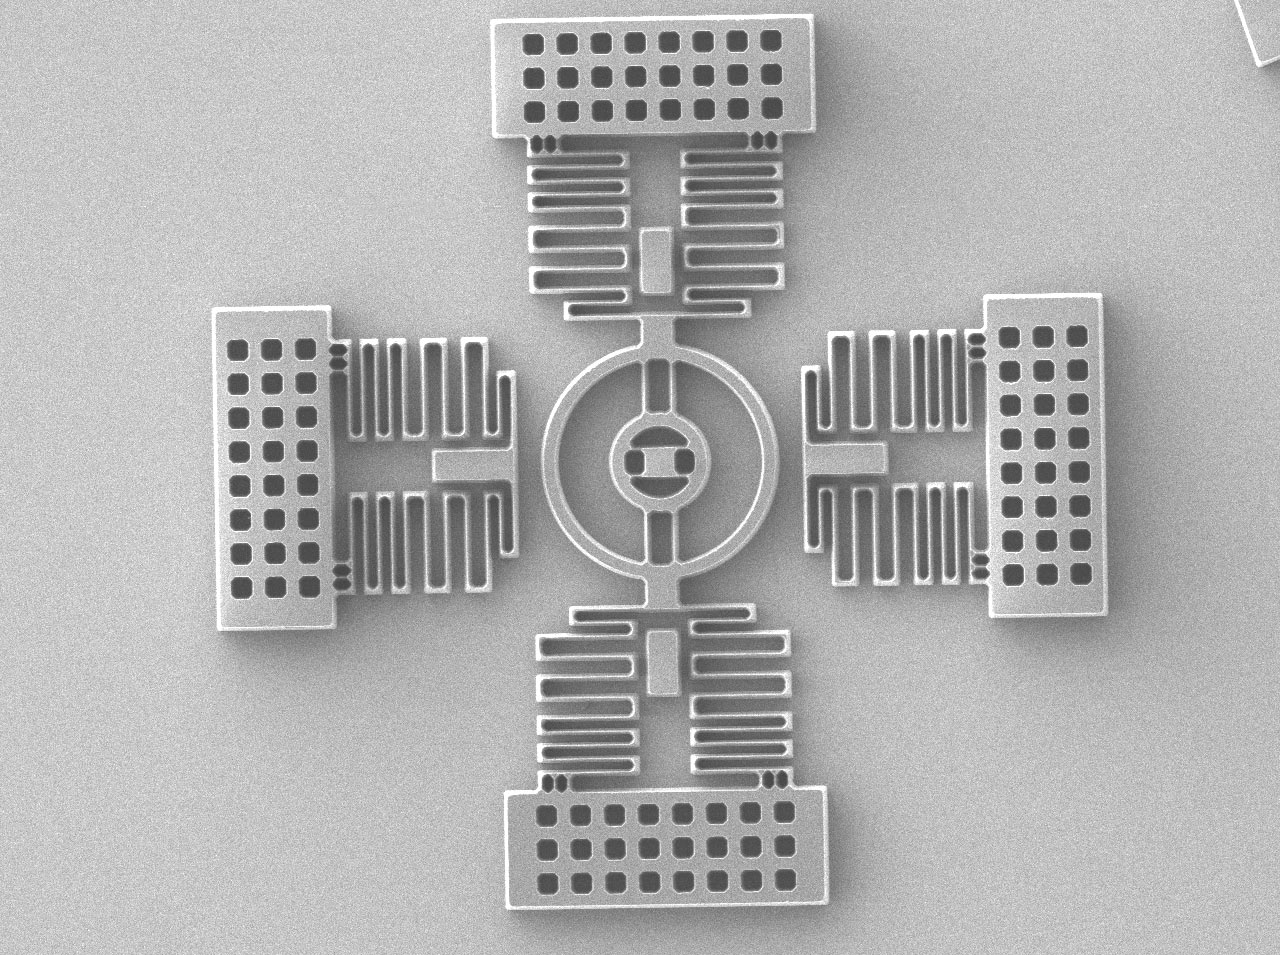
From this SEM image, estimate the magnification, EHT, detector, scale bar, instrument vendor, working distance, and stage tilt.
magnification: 0.16 K X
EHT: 2 kV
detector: SEI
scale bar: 100000 nm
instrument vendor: JEOL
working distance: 8 mm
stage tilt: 0°